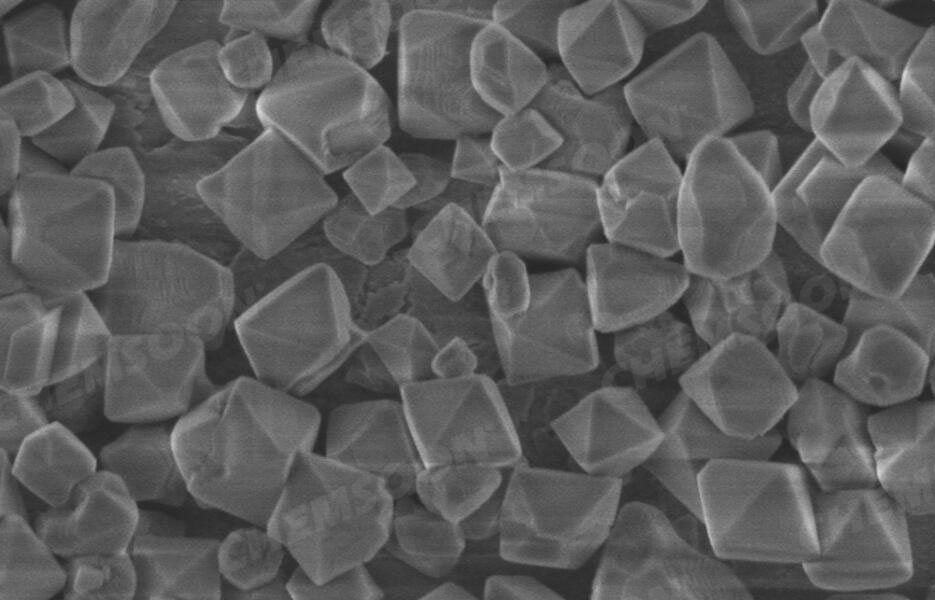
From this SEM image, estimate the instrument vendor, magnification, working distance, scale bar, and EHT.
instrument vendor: Hitachi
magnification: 40 K X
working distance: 2.6 mm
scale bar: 1000 nm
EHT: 1 kV